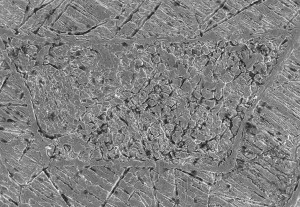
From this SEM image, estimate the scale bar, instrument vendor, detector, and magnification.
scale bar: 500000 nm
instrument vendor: Hitachi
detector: SE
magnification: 0.1 K X